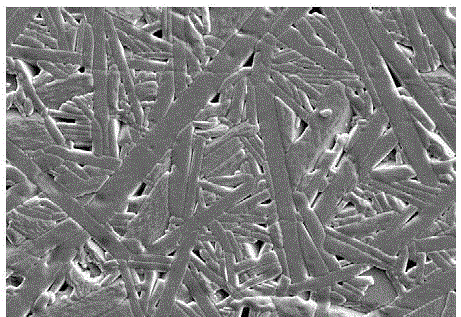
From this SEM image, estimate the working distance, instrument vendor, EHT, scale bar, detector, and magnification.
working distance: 11 mm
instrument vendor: JEOL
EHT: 20 kV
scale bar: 10000 nm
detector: SEI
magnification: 1 K X